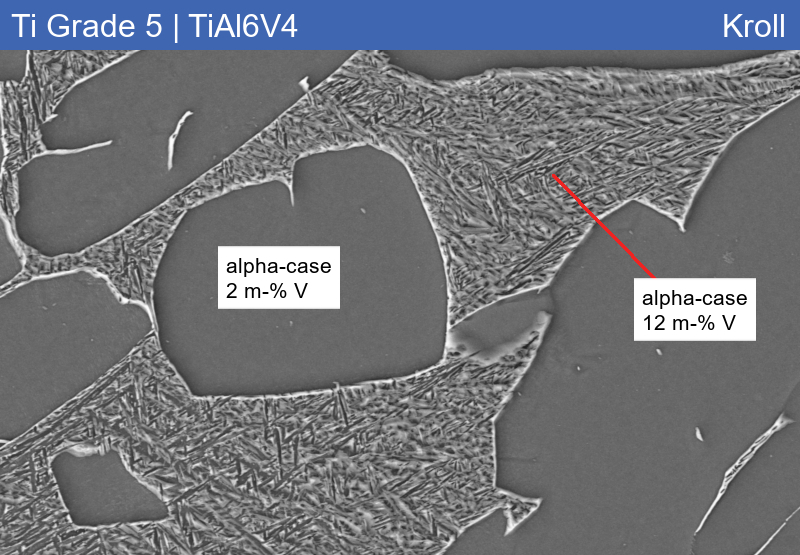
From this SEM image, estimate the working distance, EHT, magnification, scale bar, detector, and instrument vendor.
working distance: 10.1 mm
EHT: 15 kV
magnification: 2 K X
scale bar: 2000 nm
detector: SE2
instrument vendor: Zeiss